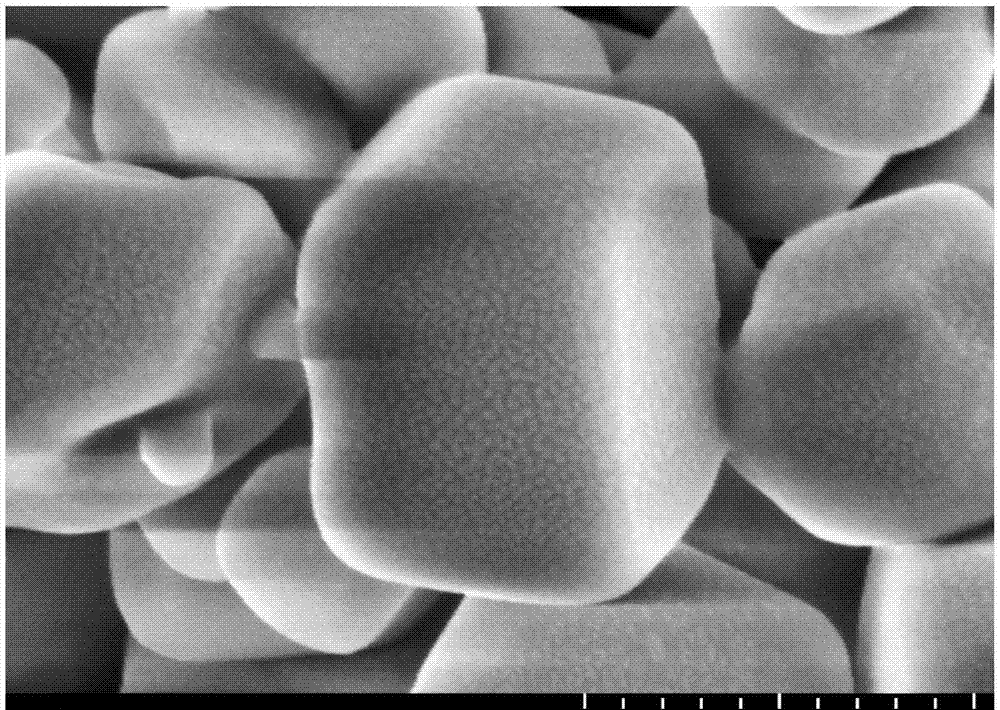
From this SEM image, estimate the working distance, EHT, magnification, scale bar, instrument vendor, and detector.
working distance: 10.5 mm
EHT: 5 kV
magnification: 50 K X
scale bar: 1000 nm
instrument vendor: Hitachi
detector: SE(UL)